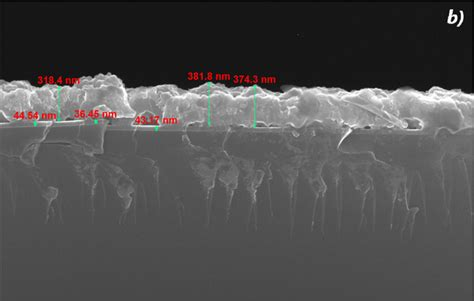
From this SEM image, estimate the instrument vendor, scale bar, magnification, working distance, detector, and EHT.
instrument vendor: FEI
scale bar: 1000 nm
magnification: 100 K X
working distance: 4.3 mm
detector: TLD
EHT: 25 kV